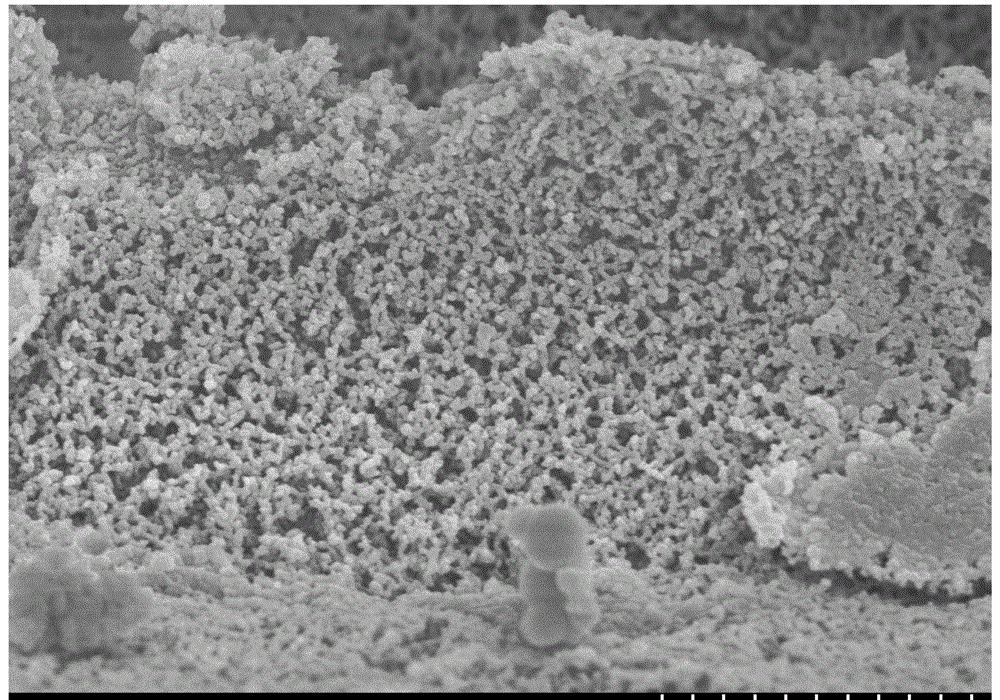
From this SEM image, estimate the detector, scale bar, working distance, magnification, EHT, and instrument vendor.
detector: SE(M)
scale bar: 2000 nm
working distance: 8.3 mm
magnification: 20 K X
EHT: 3 kV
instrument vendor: Hitachi